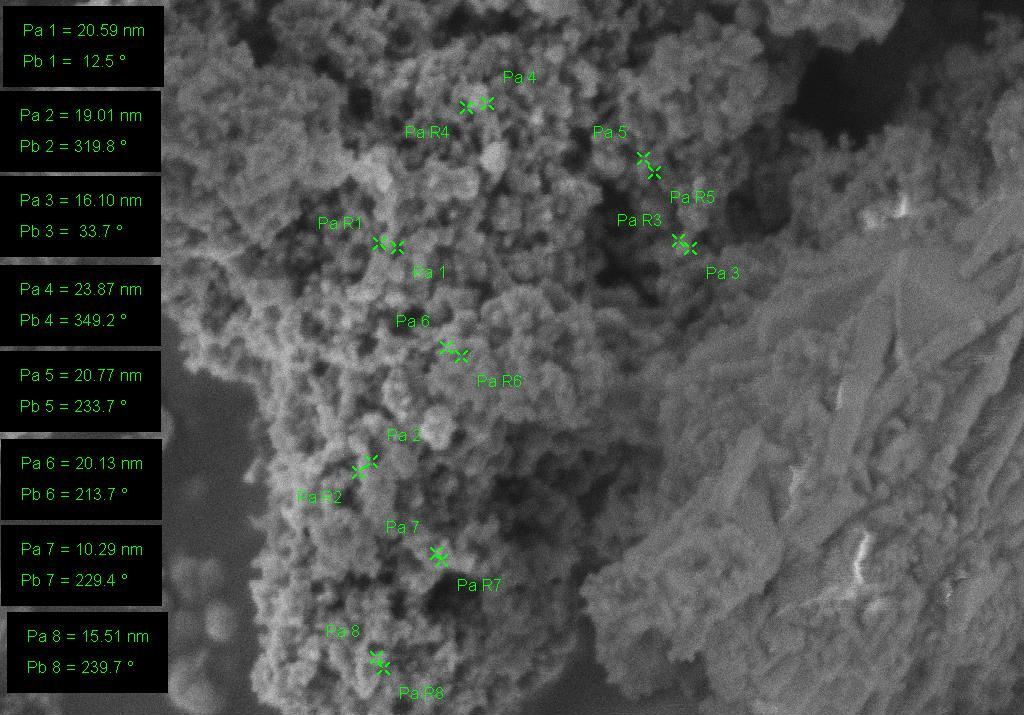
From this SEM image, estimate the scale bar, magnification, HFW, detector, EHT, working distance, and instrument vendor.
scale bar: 100 nm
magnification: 100 K X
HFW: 1.143 µm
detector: InLens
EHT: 1 kV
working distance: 2 mm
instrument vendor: Zeiss